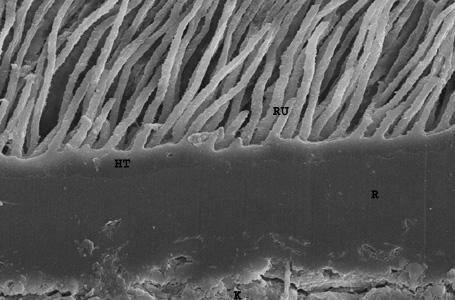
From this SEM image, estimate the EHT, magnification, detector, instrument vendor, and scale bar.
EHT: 20 kV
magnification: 1 K X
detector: SE1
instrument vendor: Zeiss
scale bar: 20000 nm